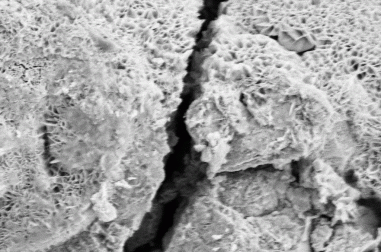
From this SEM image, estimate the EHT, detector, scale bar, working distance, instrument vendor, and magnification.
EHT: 5 kV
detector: SE2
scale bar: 2000 nm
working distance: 12.7 mm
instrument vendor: Zeiss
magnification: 40.25 K X